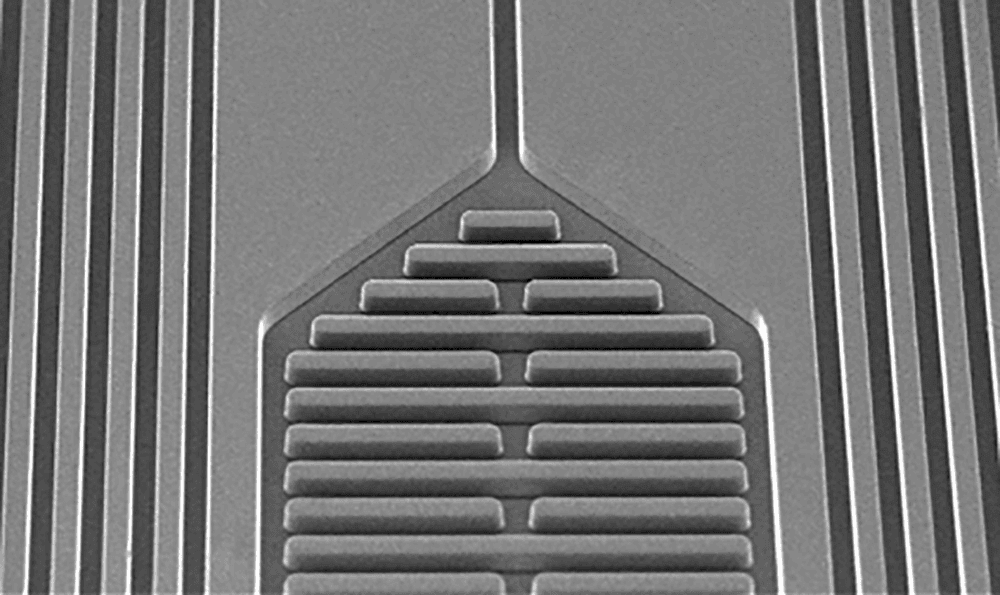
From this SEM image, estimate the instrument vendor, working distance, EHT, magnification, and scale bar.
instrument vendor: JEOL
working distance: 10.3 mm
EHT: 15 kV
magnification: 0.06 K X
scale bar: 500000 nm